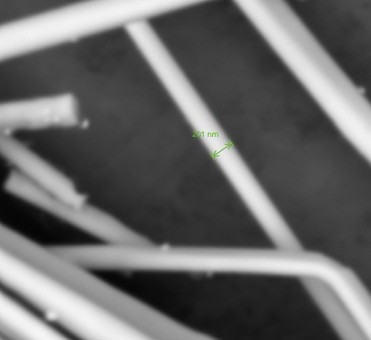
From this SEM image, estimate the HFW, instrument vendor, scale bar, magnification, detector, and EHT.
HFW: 3.19 µm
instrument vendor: other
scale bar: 1000 nm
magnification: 85 K X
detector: BSD Full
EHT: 10 kV